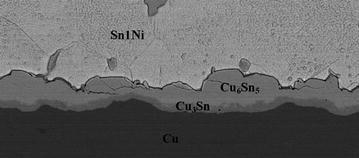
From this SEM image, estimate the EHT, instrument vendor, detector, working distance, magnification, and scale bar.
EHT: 15 kV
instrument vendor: JEOL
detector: COMPO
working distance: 14.9 mm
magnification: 1 K X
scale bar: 10000 nm